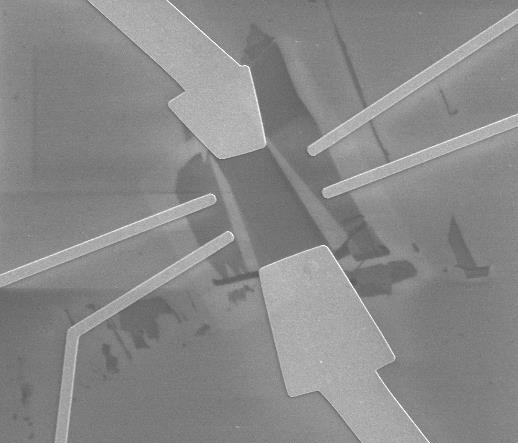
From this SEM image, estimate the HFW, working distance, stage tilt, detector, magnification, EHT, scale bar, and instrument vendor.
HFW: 51.3 µm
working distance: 3.7 mm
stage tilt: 0°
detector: ETD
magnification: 2.496 K X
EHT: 5 kV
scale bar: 20000 nm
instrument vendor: FEI